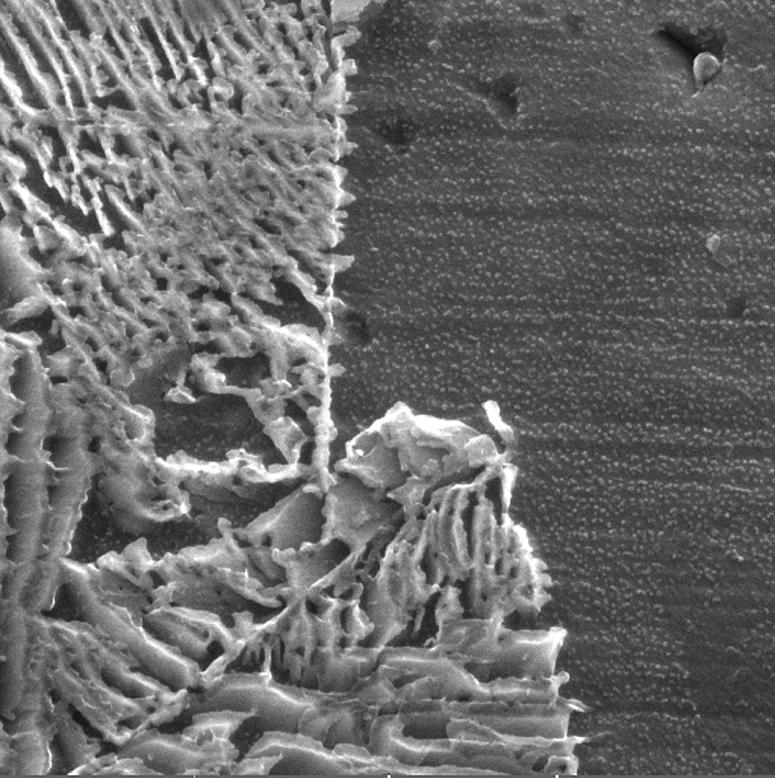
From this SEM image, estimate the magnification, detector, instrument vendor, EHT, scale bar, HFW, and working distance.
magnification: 30 K X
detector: SE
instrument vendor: Tescan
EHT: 15 kV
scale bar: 1000 nm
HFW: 4.61 µm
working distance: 12.46 mm